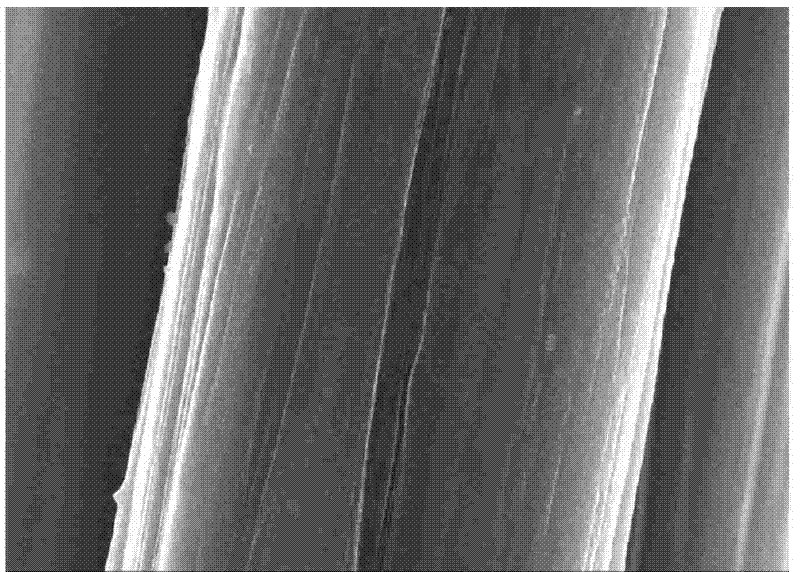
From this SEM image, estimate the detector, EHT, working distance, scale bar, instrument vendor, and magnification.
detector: SE(M,LA0)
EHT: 15 kV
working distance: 9.4 mm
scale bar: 5000 nm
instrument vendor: Hitachi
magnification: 10 K X